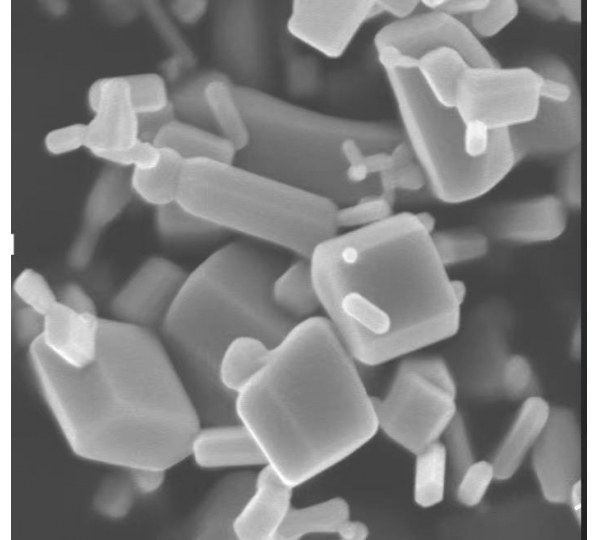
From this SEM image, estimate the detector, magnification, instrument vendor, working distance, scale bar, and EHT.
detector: SEI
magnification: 45 K X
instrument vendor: JEOL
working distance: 3 mm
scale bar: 100 nm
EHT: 5 kV